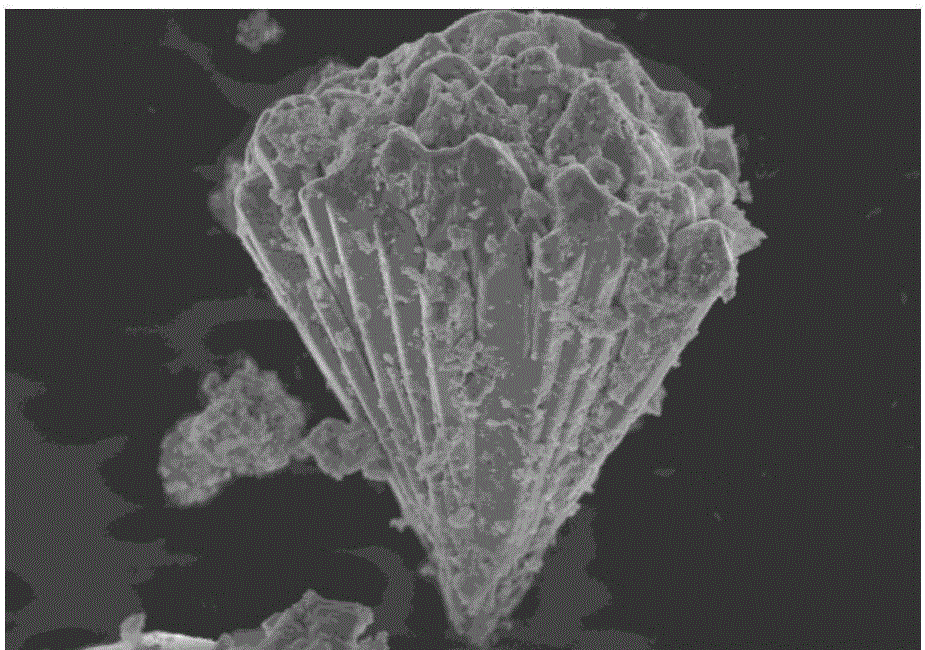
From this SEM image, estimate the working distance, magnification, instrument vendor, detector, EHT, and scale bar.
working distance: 7.2 mm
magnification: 7 K X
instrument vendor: Hitachi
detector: SE(U)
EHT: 5 kV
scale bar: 5000 nm